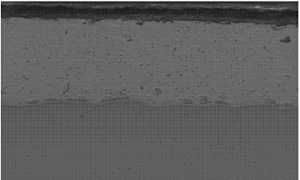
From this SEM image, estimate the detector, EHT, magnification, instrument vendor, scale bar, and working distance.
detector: BSE3D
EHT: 15 kV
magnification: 0.6 K X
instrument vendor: Hitachi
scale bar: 50000 nm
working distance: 9.5 mm